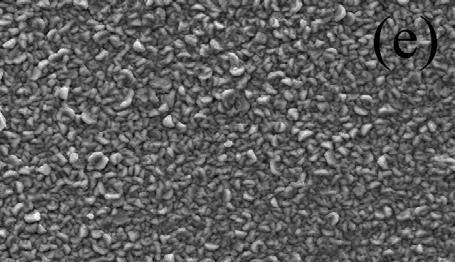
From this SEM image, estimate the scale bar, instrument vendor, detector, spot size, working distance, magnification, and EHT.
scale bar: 20000 nm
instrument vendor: FEI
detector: SE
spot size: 4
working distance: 11 mm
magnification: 1 K X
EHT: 25 kV